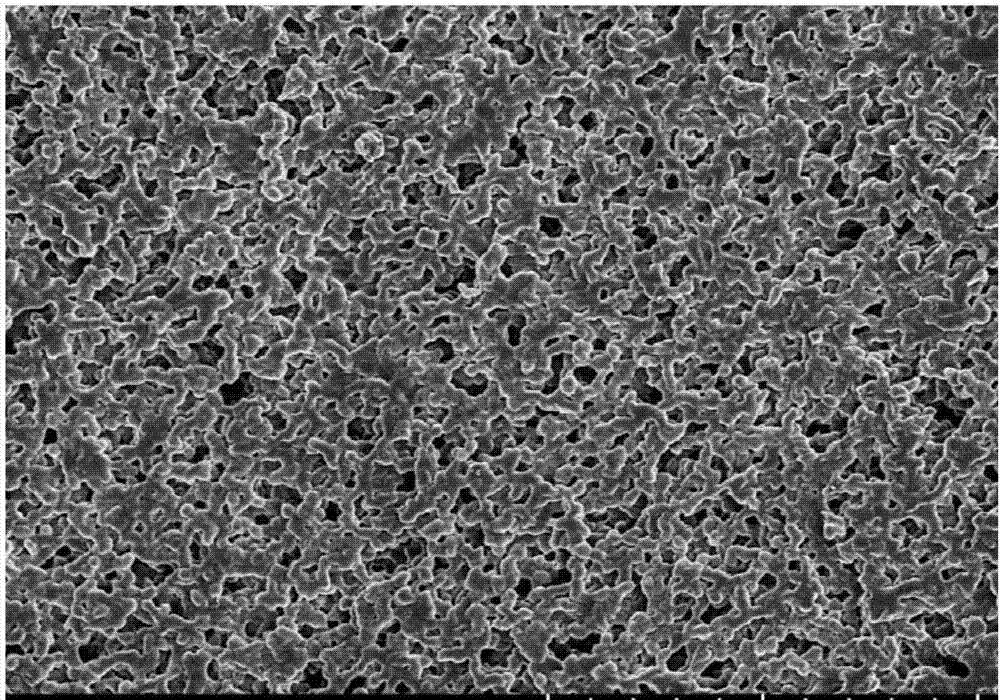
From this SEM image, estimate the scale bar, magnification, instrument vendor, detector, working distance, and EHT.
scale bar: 50000 nm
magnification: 1.1 K X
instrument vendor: Hitachi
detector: SE(UL)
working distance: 13 mm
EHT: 5 kV